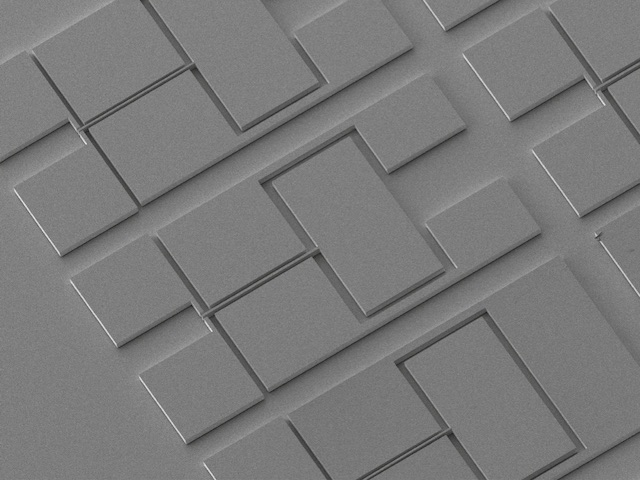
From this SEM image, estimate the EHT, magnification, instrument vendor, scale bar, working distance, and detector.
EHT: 5 kV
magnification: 0.085 K X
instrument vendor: JEOL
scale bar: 100000 nm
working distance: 20 mm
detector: LEI